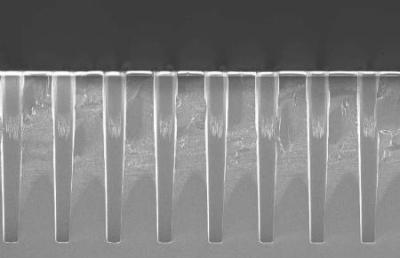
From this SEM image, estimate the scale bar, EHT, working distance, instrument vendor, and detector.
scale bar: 20000 nm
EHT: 5 kV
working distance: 5 mm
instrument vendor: Zeiss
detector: InLens